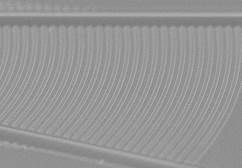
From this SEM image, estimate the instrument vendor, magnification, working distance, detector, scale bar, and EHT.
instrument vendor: Hitachi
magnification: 10 K X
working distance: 0.8 mm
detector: SE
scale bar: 5000 nm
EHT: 3 kV